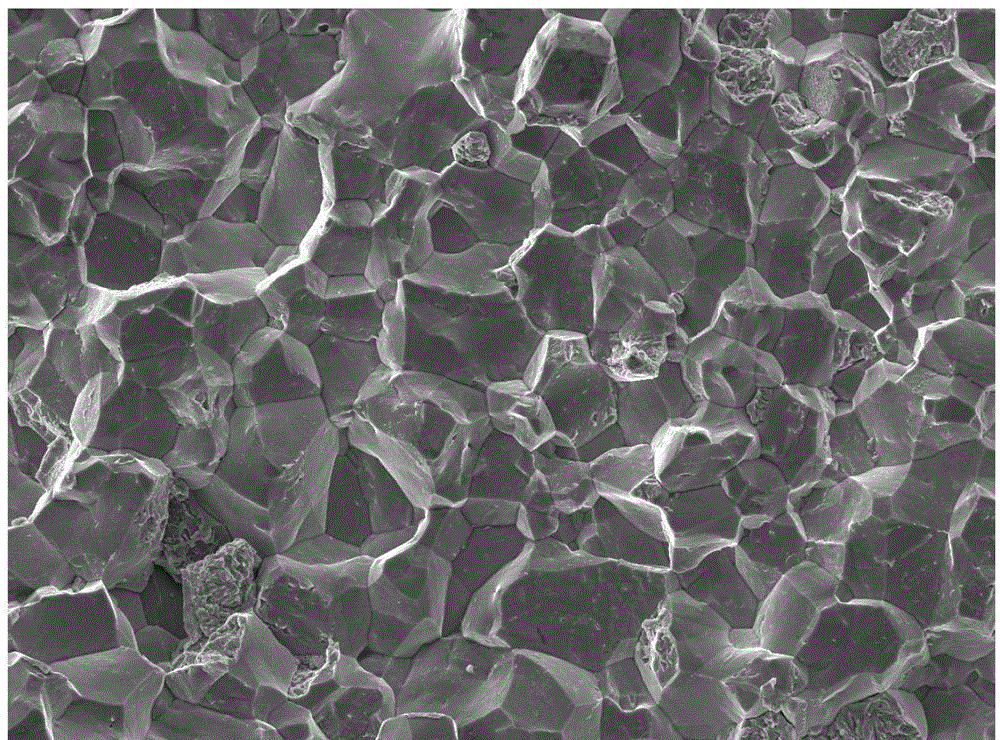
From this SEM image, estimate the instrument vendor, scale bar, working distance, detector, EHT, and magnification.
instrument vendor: JEOL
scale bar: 10000 nm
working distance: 9 mm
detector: SEI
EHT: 20 kV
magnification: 0.5 K X